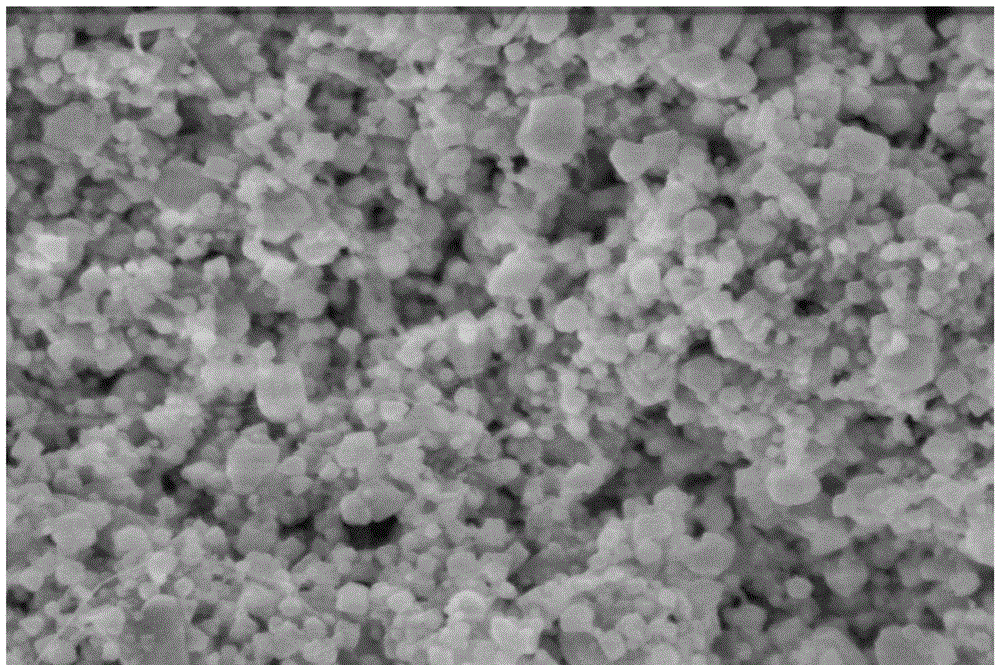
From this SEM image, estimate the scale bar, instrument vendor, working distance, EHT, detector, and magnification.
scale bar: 500 nm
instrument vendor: Hitachi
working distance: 4 mm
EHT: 3 kV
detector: SE(M)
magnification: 100 K X